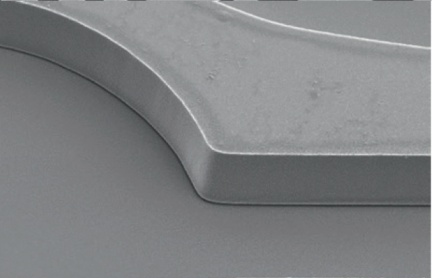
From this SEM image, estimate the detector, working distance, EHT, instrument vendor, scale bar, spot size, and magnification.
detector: SE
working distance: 7.6 mm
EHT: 5 kV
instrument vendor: FEI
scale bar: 10000 nm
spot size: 3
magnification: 2.203 K X